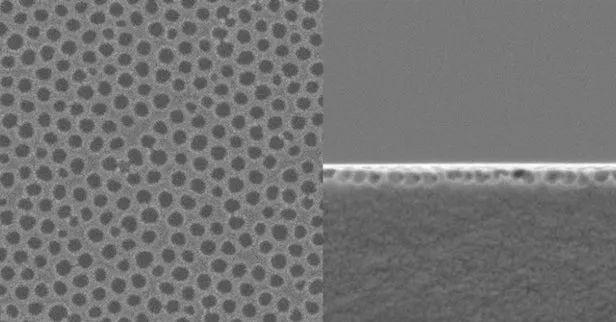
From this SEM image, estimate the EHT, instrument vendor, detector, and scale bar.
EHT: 2 kV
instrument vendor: JEOL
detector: SEI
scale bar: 1000 nm